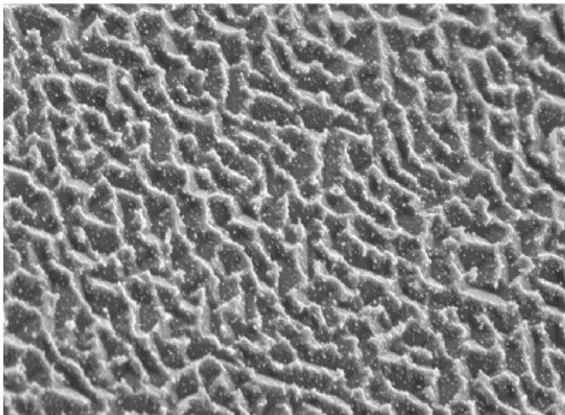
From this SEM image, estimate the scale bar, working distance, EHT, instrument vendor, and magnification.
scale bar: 1000 nm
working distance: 14 mm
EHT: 5 kV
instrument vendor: JEOL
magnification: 6 K X